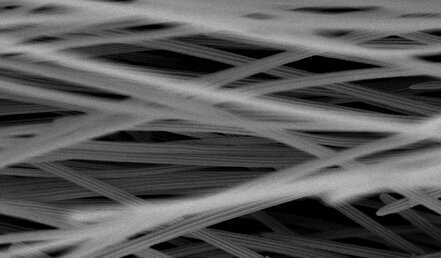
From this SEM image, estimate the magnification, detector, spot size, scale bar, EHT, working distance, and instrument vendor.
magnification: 0.8 K X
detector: SE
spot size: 3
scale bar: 20000 nm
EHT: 30 kV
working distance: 6.4 mm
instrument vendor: FEI